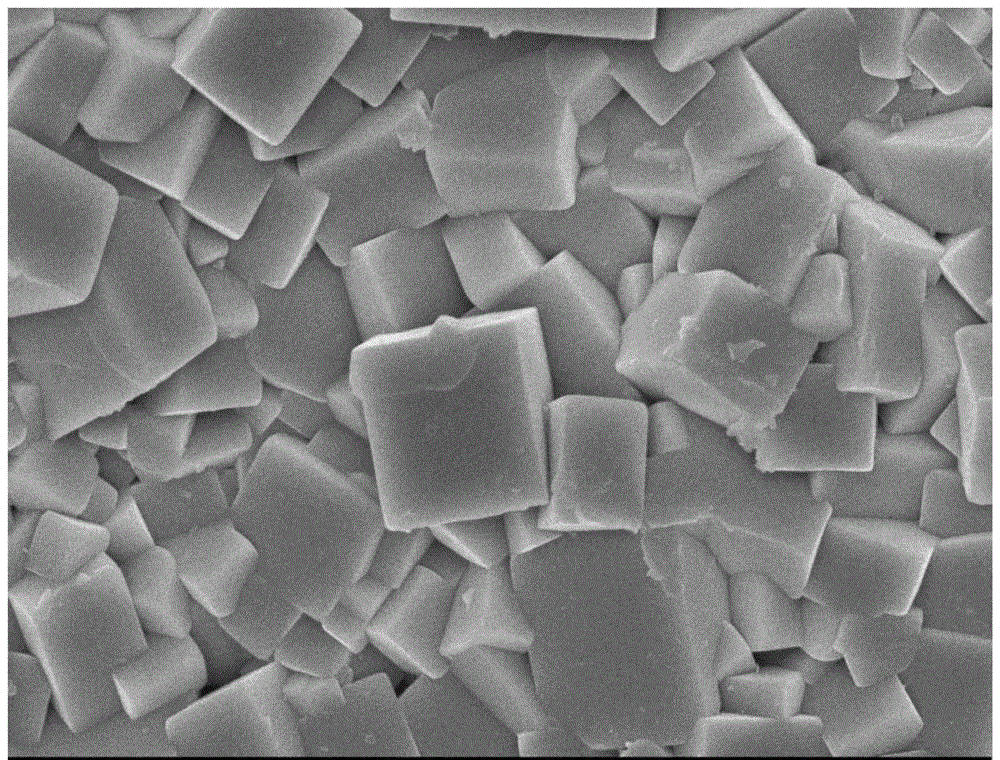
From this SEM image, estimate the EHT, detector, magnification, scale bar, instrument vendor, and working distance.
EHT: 10 kV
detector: SEI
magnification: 15 K X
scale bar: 1000 nm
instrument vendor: JEOL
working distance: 8.6 mm